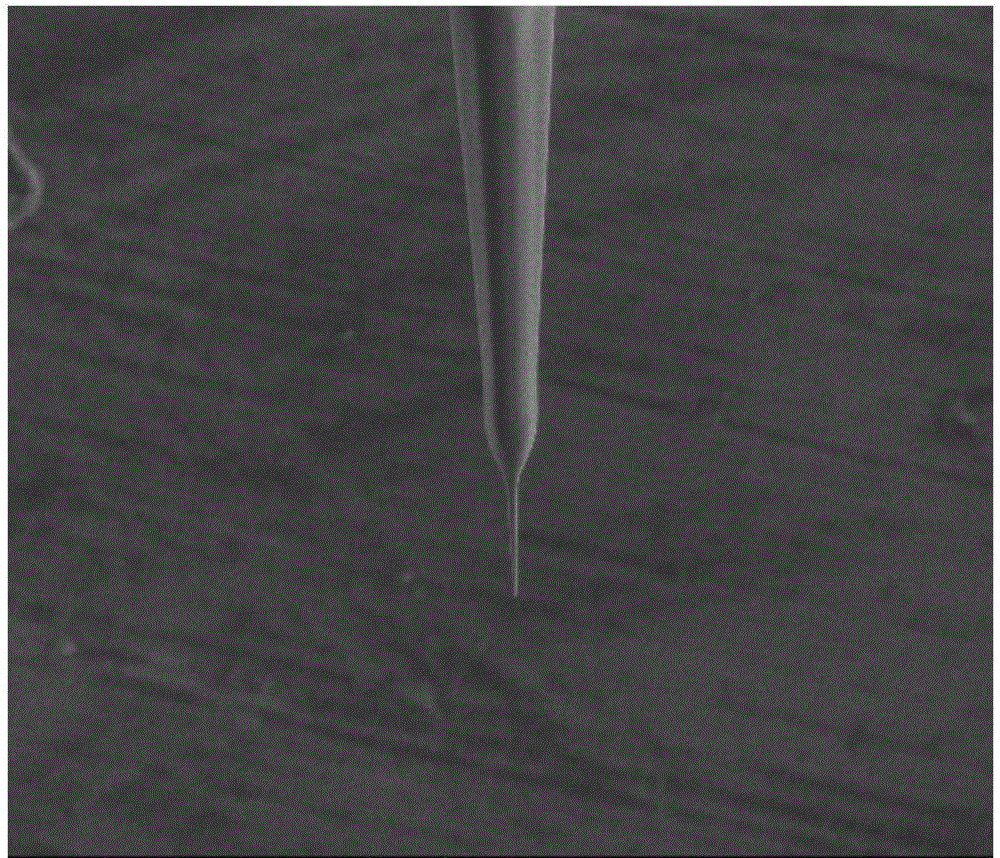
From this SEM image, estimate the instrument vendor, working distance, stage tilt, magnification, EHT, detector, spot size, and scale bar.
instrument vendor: FEI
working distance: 4.789 mm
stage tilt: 0°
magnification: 2 K X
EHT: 5 kV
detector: SED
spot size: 2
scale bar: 20000 nm